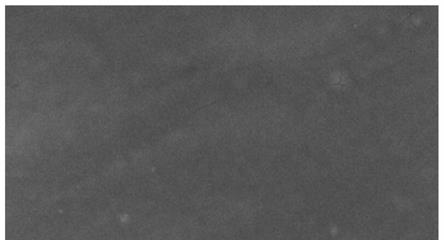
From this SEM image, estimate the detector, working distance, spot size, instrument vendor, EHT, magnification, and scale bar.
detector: ETD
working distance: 13.6 mm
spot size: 4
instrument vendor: FEI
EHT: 20 kV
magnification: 1.2 K X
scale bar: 50000 nm